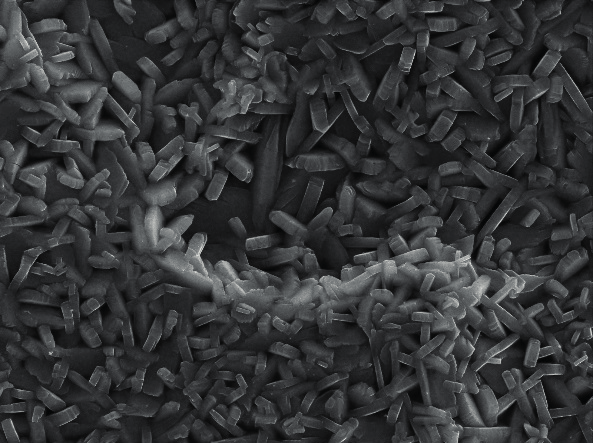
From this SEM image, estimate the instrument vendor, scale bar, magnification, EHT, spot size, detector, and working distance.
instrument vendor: other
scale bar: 2000 nm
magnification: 5 K X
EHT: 30 kV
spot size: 1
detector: SEI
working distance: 7.54 mm